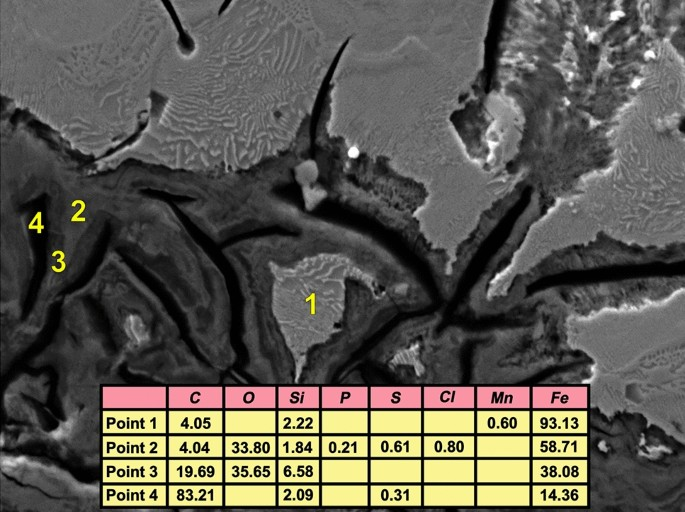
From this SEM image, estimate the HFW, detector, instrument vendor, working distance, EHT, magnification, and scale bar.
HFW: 83.9 µm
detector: SE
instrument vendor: Tescan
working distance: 15.27 mm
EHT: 20 kV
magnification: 3.3 K X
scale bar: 20000 nm